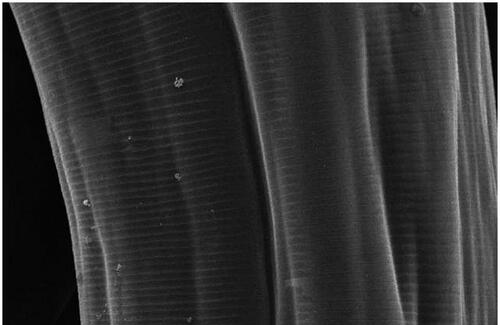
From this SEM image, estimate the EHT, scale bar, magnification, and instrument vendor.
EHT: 20 kV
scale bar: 200000 nm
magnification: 0.09 K X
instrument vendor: JEOL